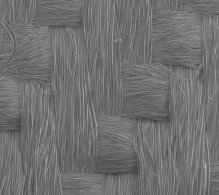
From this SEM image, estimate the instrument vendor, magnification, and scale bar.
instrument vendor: JEOL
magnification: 0.1 K X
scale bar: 100000 nm